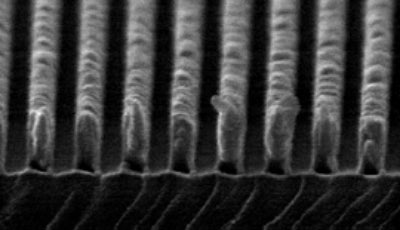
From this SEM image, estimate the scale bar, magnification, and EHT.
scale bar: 100 nm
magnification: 100 K X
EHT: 25 kV